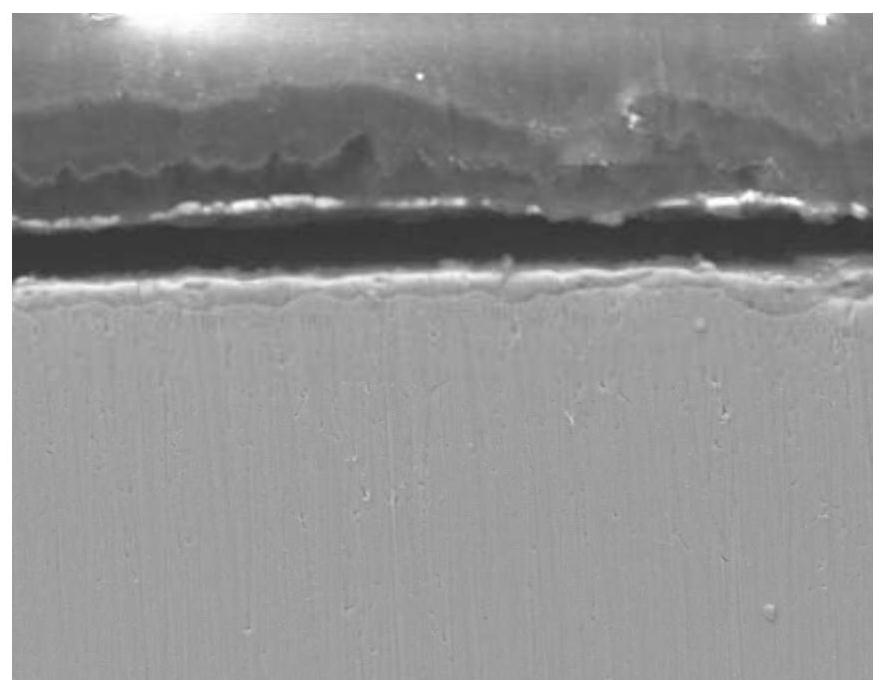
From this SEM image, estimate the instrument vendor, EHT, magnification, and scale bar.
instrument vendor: JEOL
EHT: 20 kV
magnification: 1 K X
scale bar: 10000 nm